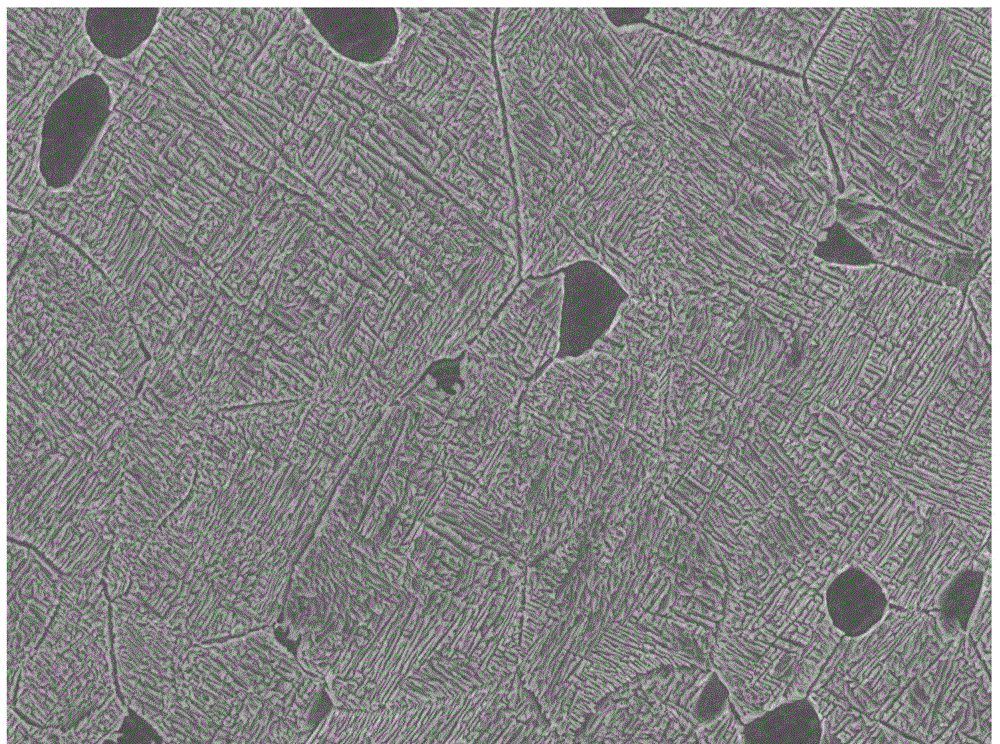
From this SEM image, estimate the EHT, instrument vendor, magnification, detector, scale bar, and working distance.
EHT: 5 kV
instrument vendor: JEOL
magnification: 5 K X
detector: SEI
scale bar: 1000 nm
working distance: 8.7 mm